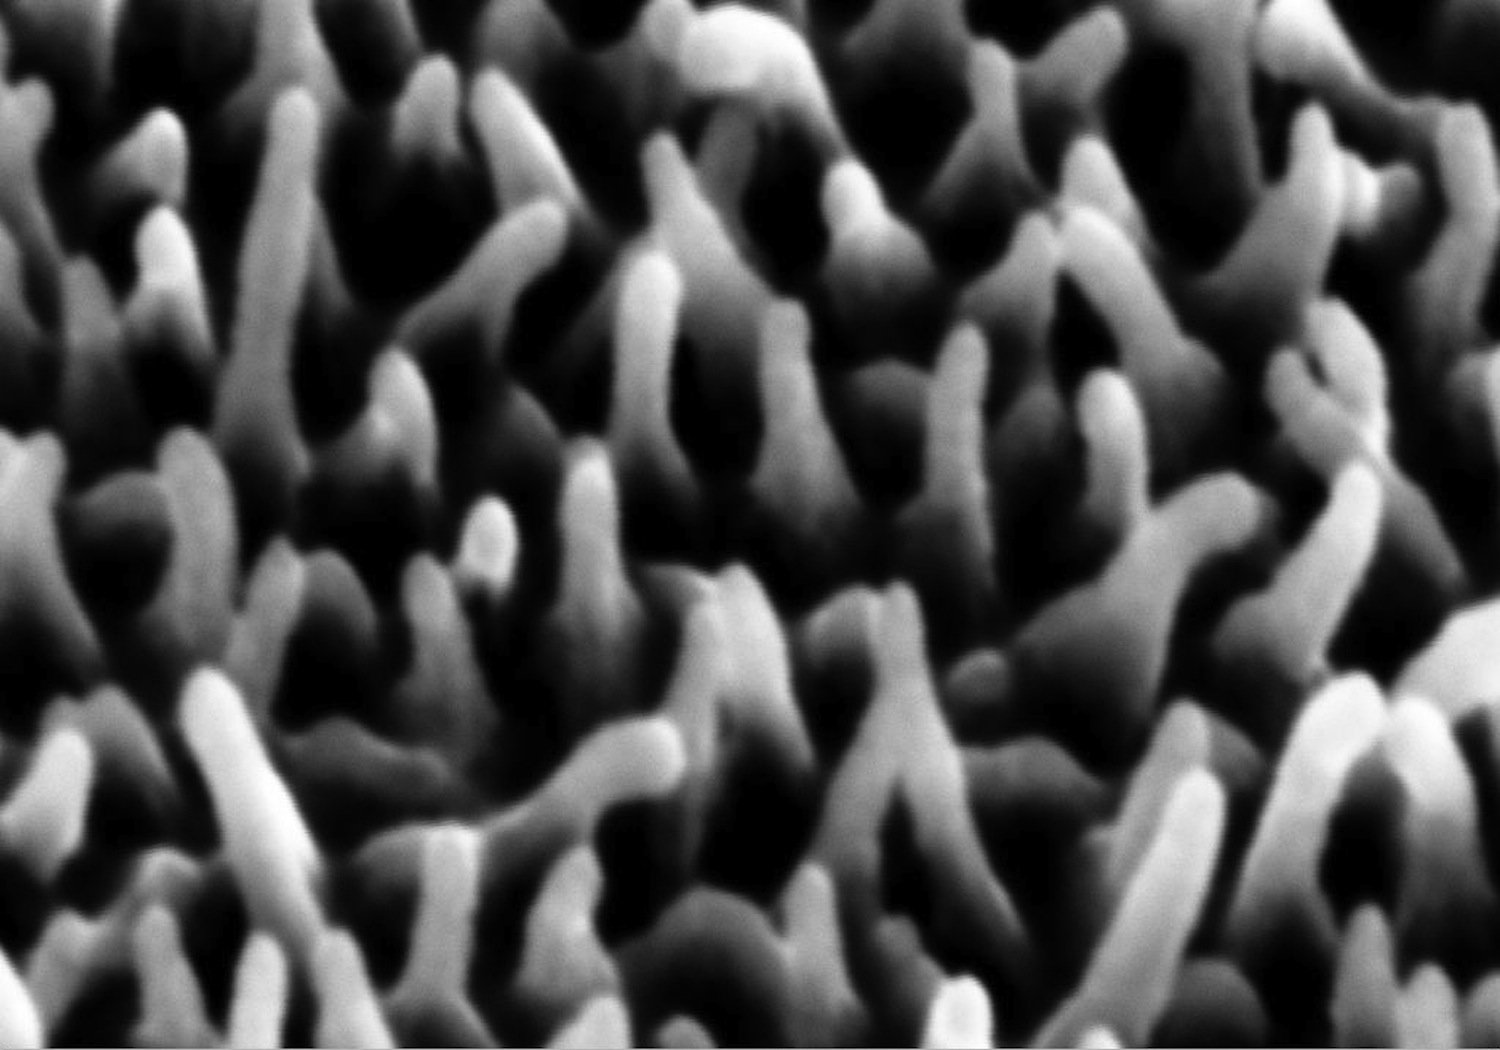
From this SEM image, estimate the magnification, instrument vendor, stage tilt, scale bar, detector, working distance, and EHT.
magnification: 60 K X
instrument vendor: Zeiss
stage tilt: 45°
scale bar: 100 nm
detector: SE2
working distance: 18.5 mm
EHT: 15 kV